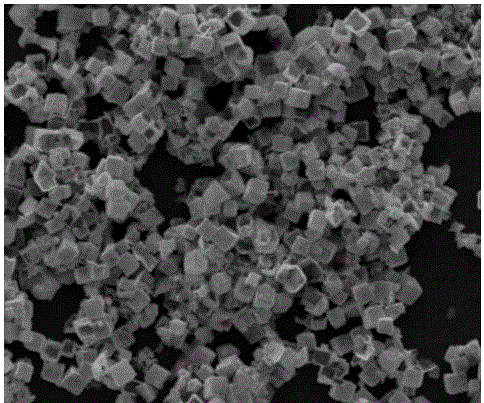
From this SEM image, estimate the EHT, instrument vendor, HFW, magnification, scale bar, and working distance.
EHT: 10 kV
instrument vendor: FEI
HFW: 24.9 µm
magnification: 12 K X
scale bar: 10000 nm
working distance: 11.3 mm